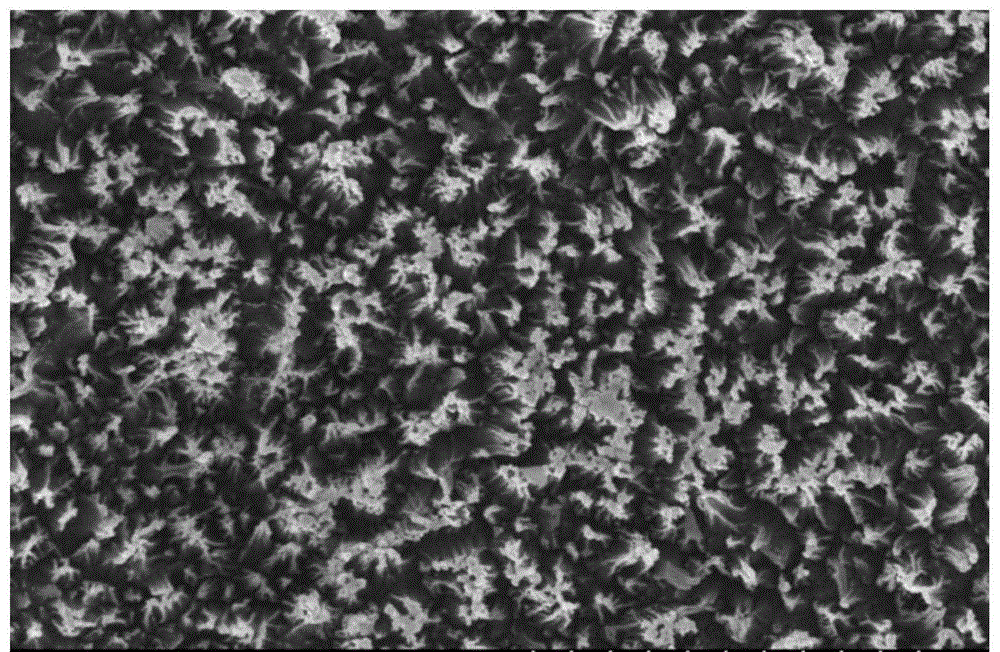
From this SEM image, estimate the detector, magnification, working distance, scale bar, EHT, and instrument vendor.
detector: SE(M)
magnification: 25 K X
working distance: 7.9 mm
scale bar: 2000 nm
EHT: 10 kV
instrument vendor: Hitachi